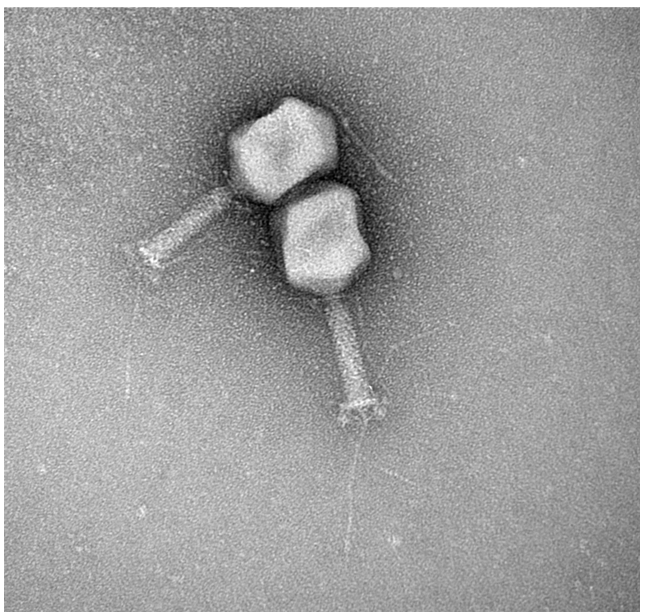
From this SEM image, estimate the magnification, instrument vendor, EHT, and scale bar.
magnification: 180 K X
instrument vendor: FEI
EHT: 120 kV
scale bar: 50 nm